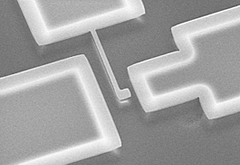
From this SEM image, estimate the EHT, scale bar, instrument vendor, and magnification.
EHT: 20 kV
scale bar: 30000 nm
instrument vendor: Hitachi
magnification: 1.5 K X